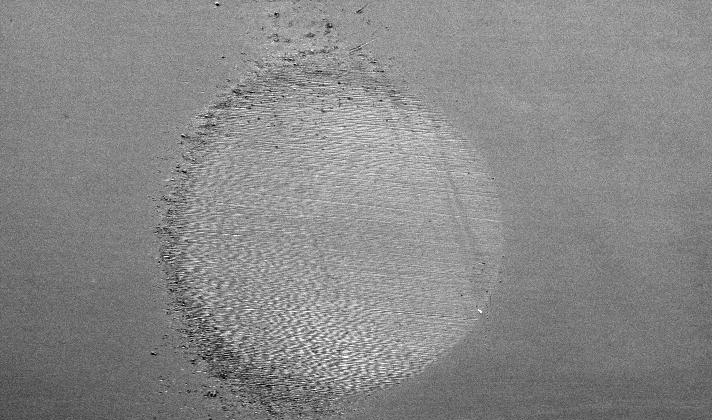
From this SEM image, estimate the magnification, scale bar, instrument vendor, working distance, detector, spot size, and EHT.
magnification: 0.04 K X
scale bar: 500000 nm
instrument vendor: FEI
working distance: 12 mm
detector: SE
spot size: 6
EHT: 20 kV